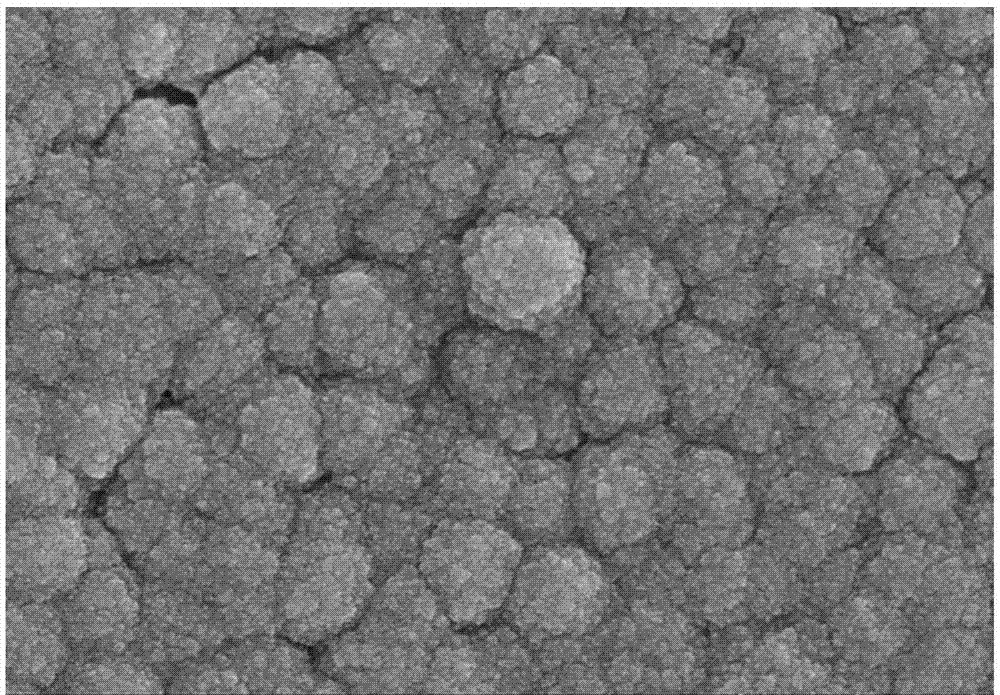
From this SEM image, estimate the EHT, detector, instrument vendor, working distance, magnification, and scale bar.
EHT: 5 kV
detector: SE(UL)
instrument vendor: Hitachi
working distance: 9.5 mm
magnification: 30 K X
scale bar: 1000 nm